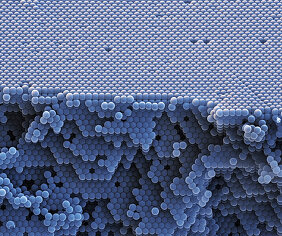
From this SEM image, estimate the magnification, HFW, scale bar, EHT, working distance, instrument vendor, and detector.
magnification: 20 K X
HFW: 7.5 µm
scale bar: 3000 nm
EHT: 7 kV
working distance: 4.8 mm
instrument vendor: FEI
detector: ETD